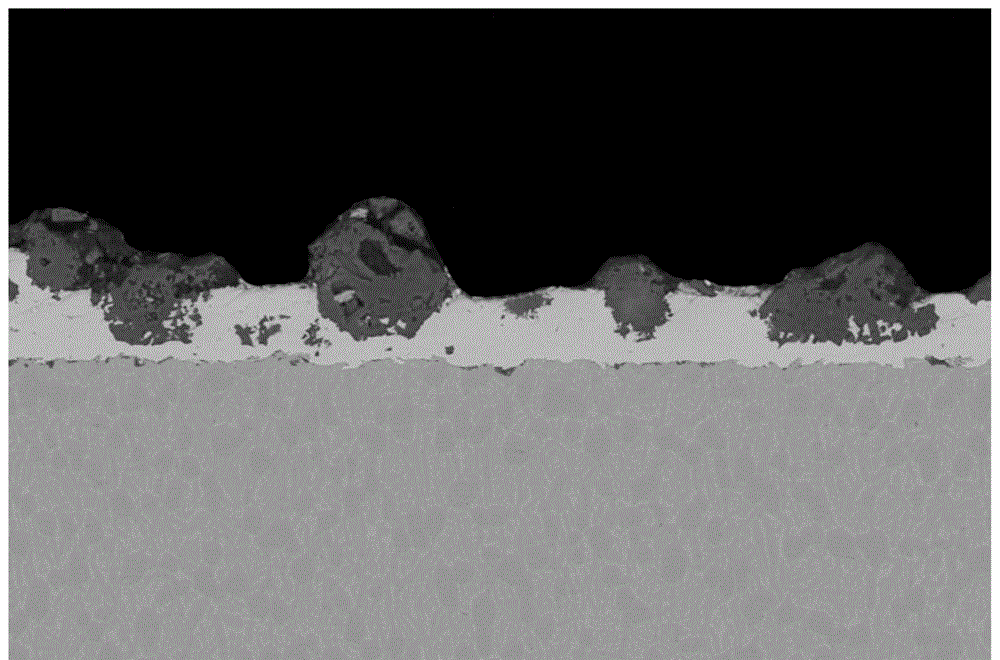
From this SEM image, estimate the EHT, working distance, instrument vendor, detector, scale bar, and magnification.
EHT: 20 kV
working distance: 12.3 mm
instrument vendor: FEI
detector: CBS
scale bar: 100000 nm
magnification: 0.5 K X